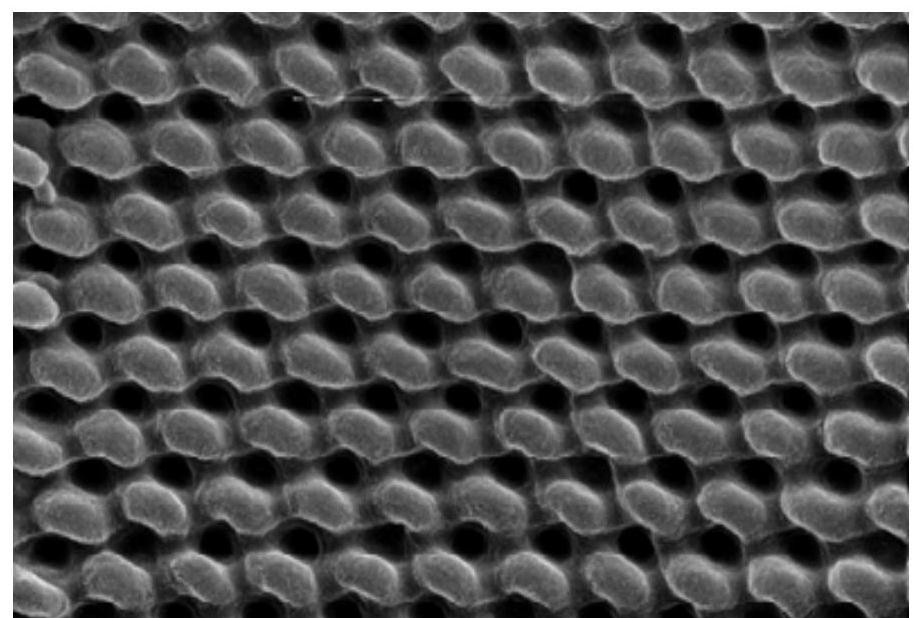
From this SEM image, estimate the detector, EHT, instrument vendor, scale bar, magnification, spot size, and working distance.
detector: TLD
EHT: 10 kV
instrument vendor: FEI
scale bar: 500 nm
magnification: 100 K X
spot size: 3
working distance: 6.4 mm